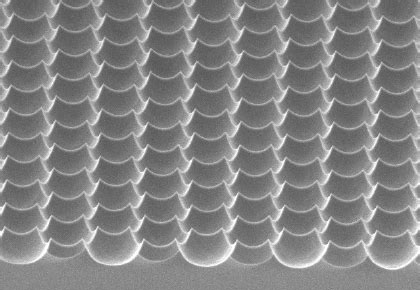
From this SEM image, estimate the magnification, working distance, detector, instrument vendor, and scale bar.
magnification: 2 K X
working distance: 11.4 mm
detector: ISE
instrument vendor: Hitachi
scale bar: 20000 nm